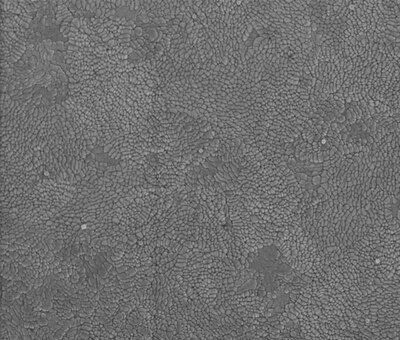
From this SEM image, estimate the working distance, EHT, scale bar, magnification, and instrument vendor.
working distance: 1.5 mm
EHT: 1 kV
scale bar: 500 nm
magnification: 159.382 K X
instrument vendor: FEI